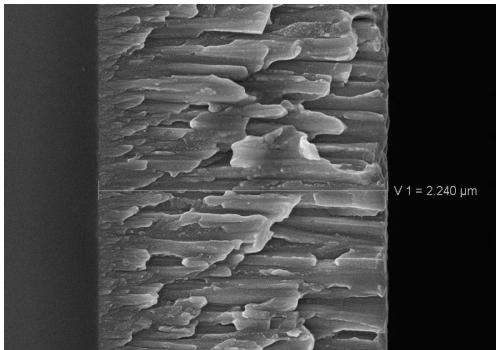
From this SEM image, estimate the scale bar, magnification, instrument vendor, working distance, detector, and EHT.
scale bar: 200 nm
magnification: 30 K X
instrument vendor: Zeiss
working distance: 7.3 mm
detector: InLens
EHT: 8 kV